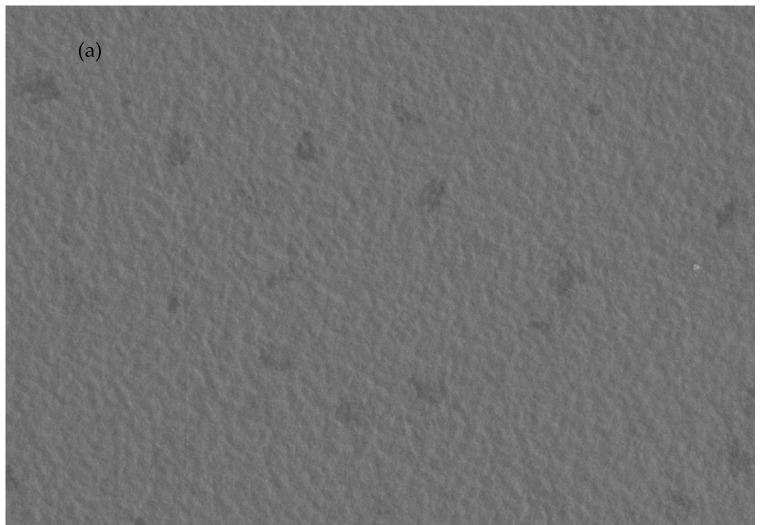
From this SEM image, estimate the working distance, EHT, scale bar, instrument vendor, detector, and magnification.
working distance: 12.9 mm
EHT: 2 kV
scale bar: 50000 nm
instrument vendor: Hitachi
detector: SE(L)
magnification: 1 K X